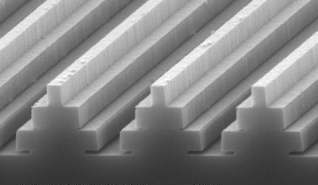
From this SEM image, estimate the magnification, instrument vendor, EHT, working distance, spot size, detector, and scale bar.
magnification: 20 K X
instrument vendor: FEI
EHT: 10 kV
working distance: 10.5 mm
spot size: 3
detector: SE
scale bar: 1000 nm